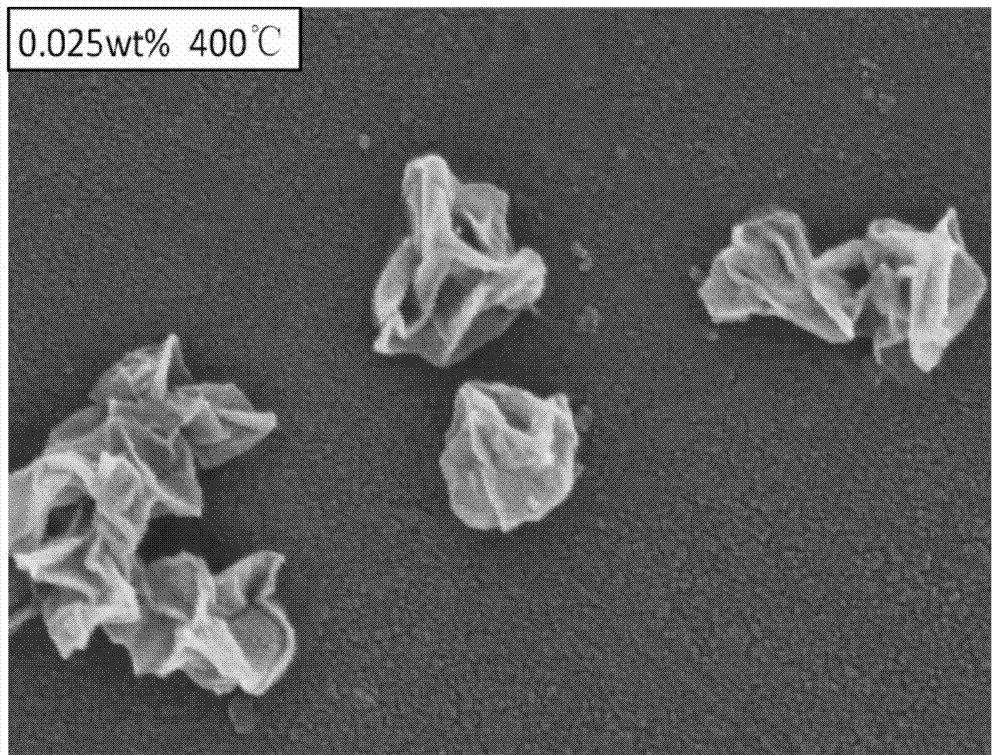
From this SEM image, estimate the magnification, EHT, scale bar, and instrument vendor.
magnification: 100 K X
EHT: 5 kV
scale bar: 500 nm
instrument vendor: Hitachi